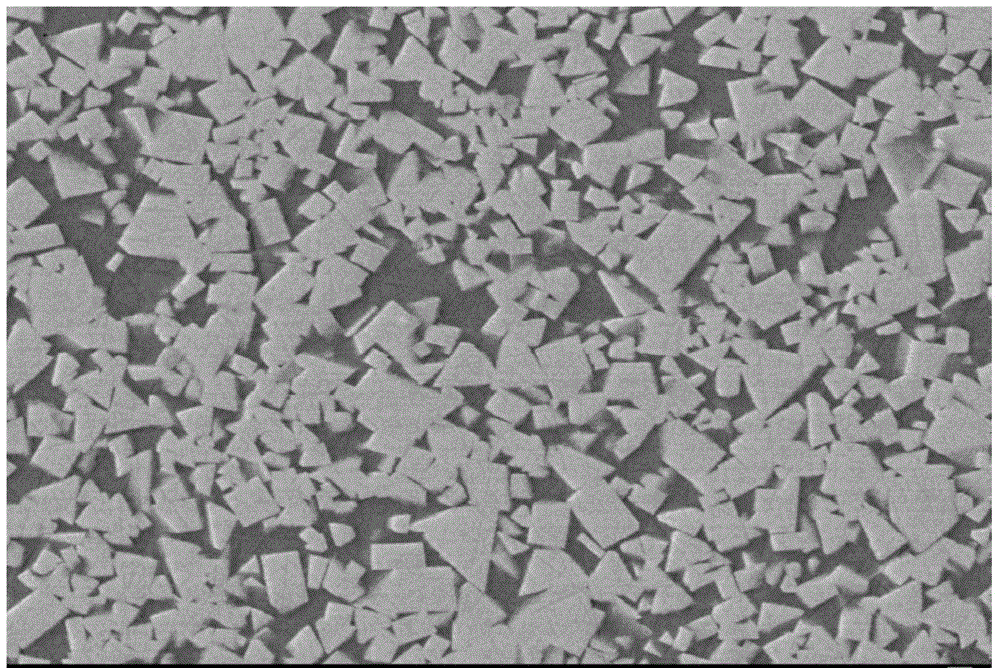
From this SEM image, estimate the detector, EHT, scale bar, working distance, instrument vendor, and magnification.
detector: LEI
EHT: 15 kV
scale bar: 1000 nm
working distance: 14.4 mm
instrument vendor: JEOL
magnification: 3 K X